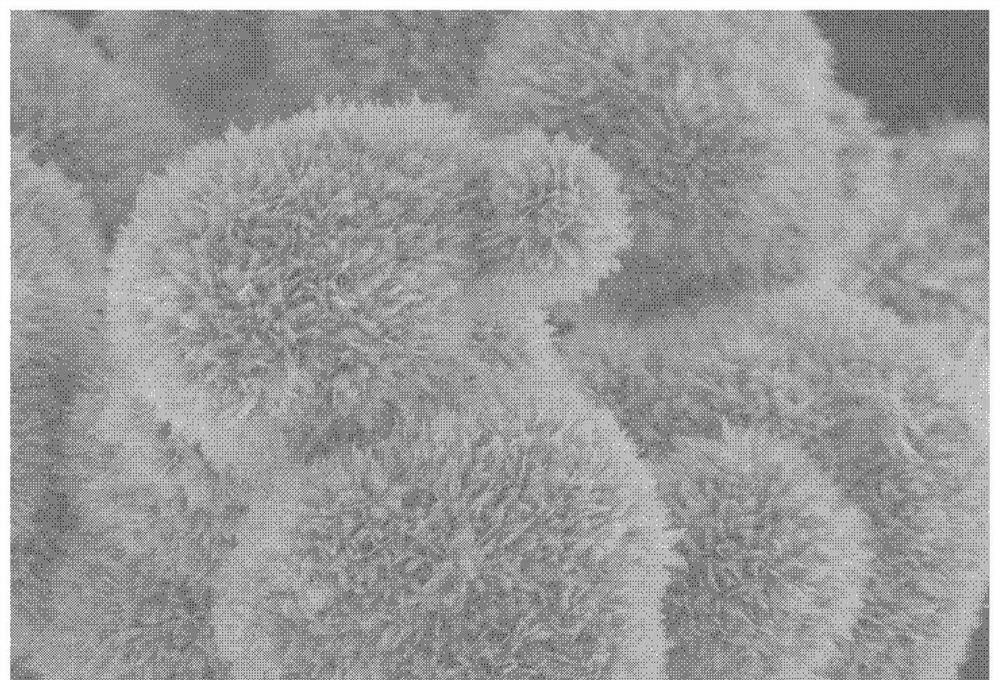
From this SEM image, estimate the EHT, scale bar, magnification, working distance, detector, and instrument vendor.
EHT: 3 kV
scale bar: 3000 nm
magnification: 18 K X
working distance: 9.2 mm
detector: SE(U)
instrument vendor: Hitachi